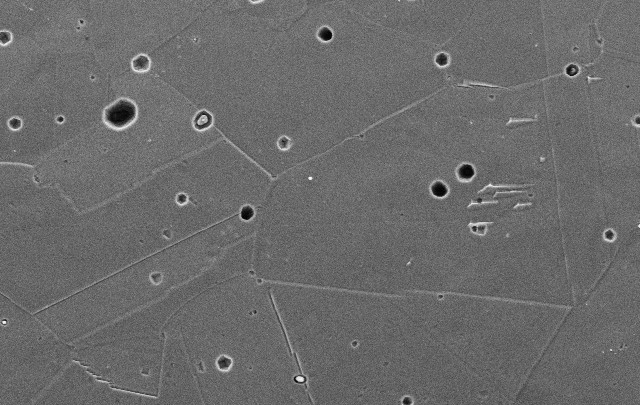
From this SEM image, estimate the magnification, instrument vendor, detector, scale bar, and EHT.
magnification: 1 K X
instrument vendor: JEOL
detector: SEI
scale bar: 20000 nm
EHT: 15 kV